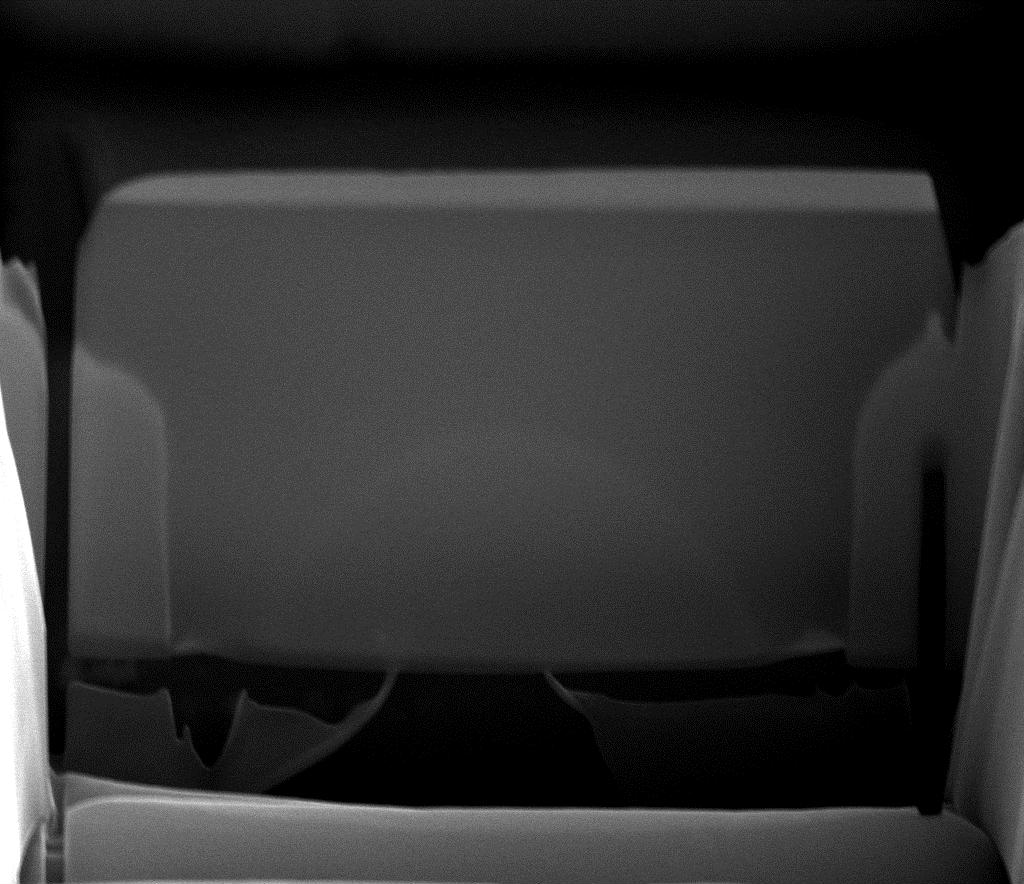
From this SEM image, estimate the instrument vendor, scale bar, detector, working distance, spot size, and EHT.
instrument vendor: FEI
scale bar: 10000 nm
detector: ETD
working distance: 9.9 mm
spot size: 4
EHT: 20 kV